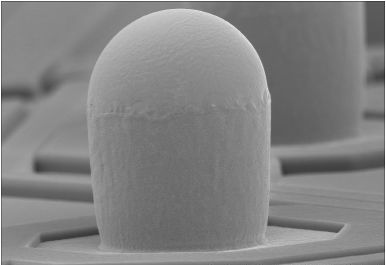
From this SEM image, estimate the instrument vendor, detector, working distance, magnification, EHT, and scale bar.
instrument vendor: Hitachi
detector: SE(M)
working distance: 7.5 mm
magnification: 1.8 K X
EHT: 15 kV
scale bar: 30000 nm